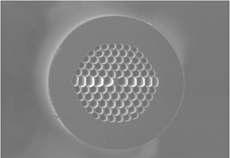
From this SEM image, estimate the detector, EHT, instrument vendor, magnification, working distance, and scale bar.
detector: SE(M)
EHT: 10 kV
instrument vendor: Hitachi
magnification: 0.6 K X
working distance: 12.6 mm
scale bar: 50000 nm